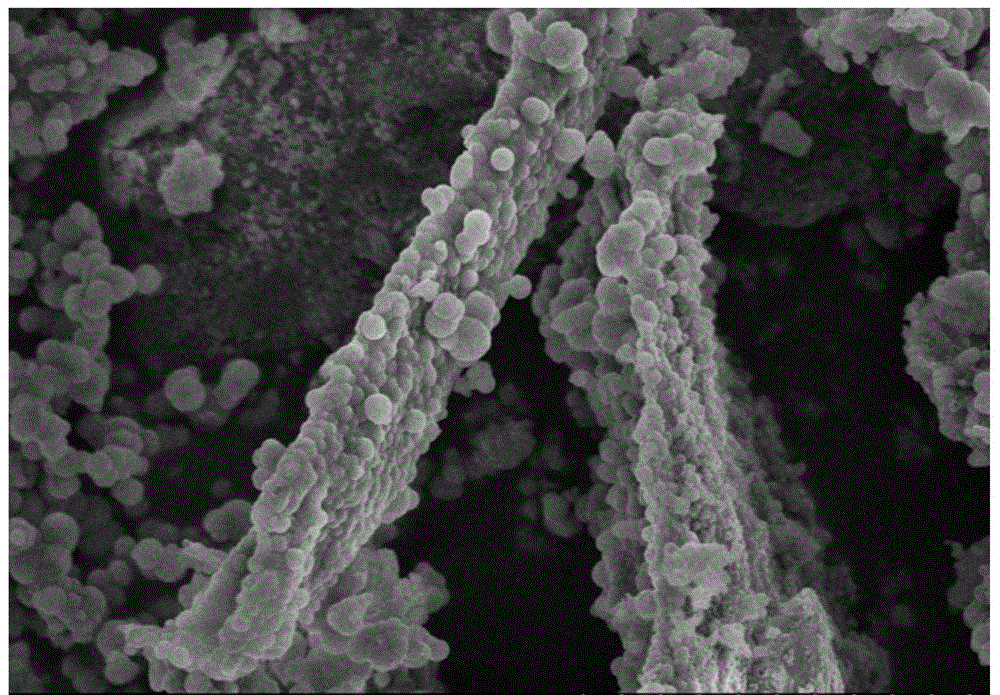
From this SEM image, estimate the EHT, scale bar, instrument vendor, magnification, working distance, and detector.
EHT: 5 kV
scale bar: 5000 nm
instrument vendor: Hitachi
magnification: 10 K X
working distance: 7.3 mm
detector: SE(M)